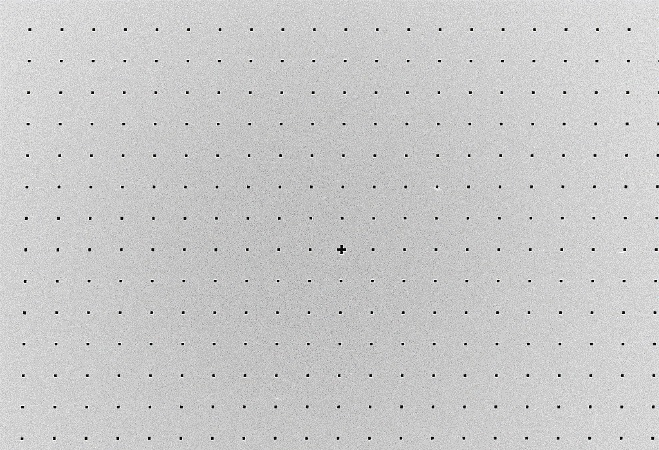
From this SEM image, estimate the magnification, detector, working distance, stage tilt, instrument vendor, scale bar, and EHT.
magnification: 0.182 K X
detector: InLens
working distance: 4.1 mm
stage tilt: -1°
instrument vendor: Zeiss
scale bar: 20000 nm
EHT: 5 kV